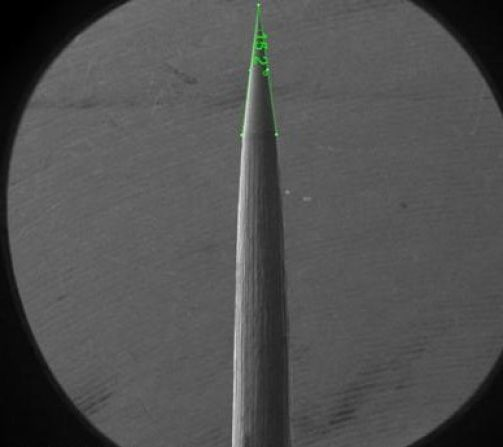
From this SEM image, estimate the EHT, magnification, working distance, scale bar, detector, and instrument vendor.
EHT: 2 kV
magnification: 0.06 K X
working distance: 5.3 mm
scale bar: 1000000 nm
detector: ETD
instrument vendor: FEI